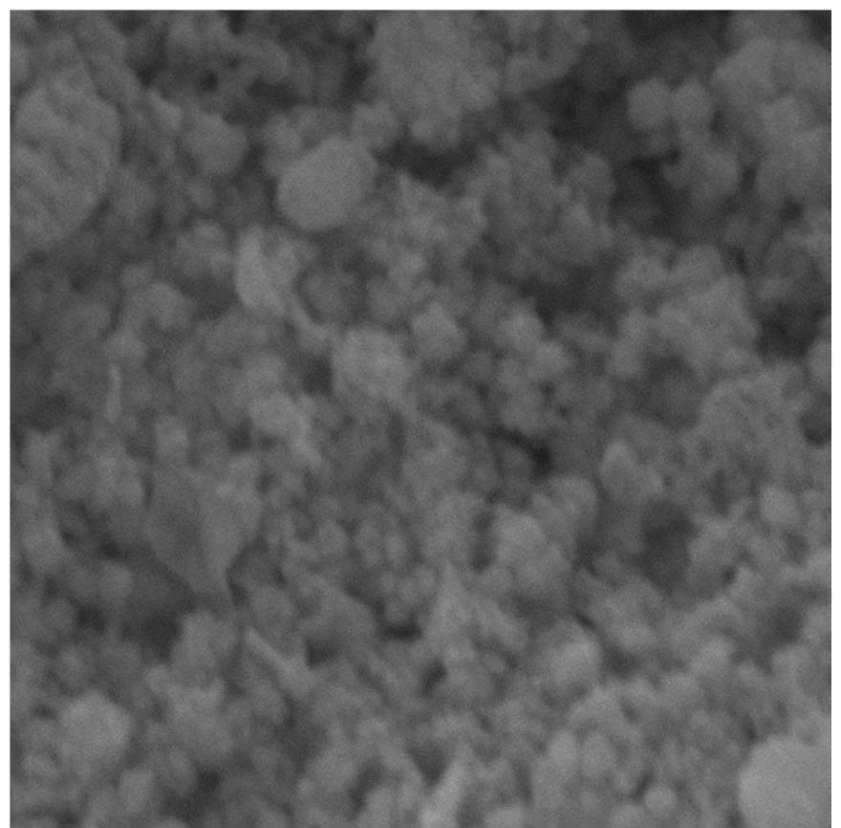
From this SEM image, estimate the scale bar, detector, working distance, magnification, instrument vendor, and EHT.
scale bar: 1000 nm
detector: SE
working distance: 3.31 mm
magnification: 49.4 K X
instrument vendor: Tescan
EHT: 20 kV